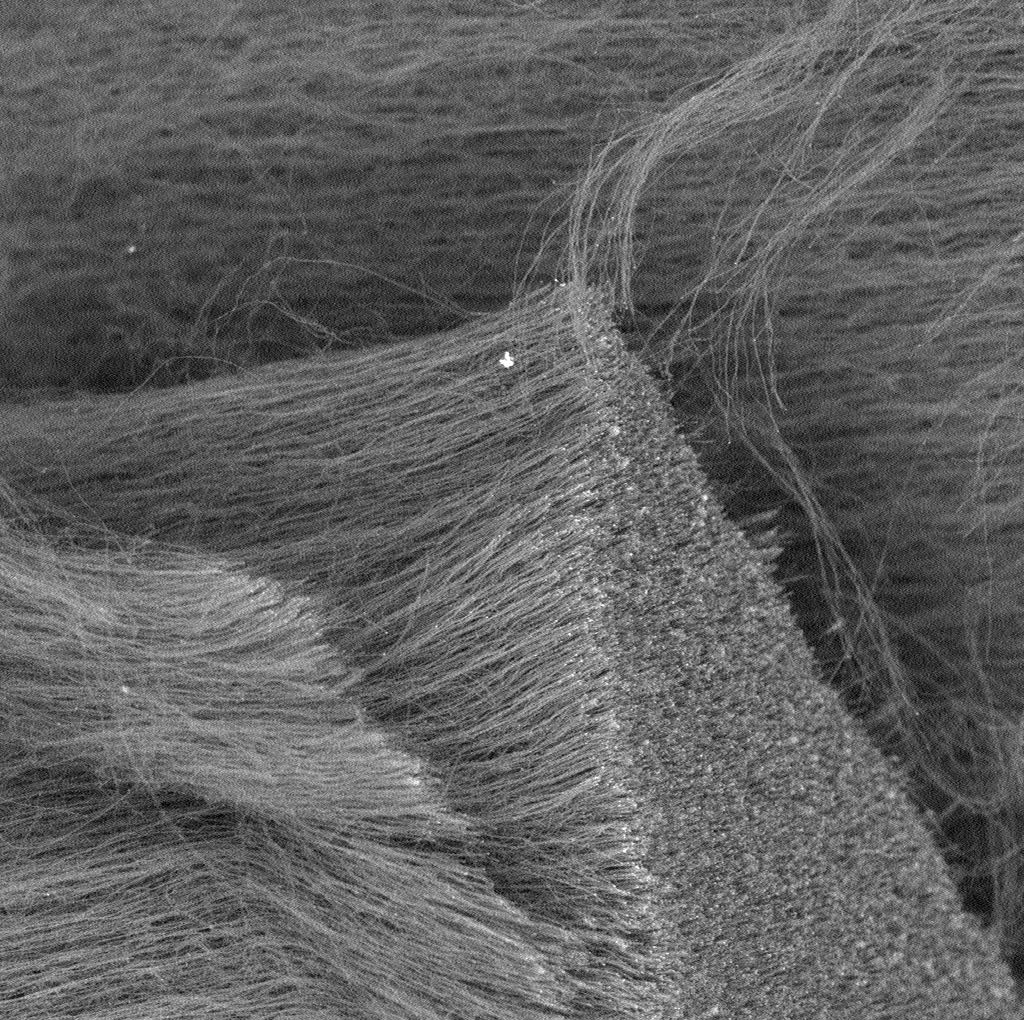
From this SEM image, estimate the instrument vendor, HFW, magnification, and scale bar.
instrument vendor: other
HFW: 169 µm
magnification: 1.18 K X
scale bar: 80000 nm